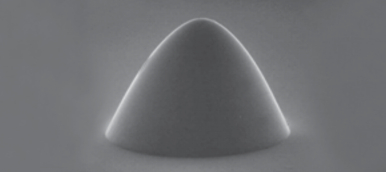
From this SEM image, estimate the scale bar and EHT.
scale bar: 10000 nm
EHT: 20 kV